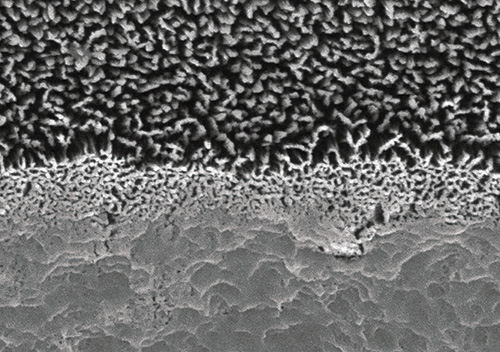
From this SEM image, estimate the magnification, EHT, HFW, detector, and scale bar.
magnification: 20 K X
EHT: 5 kV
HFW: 6 µm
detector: A+B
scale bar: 1000 nm